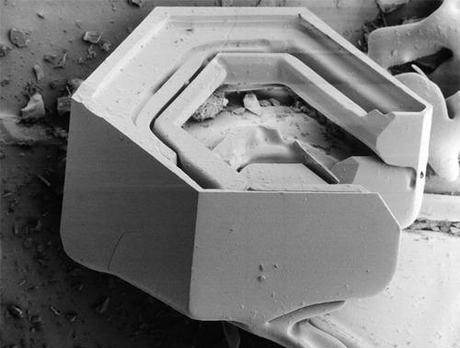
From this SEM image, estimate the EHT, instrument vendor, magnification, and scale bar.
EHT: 2 kV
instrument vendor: JEOL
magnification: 0.04 K X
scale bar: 750000 nm